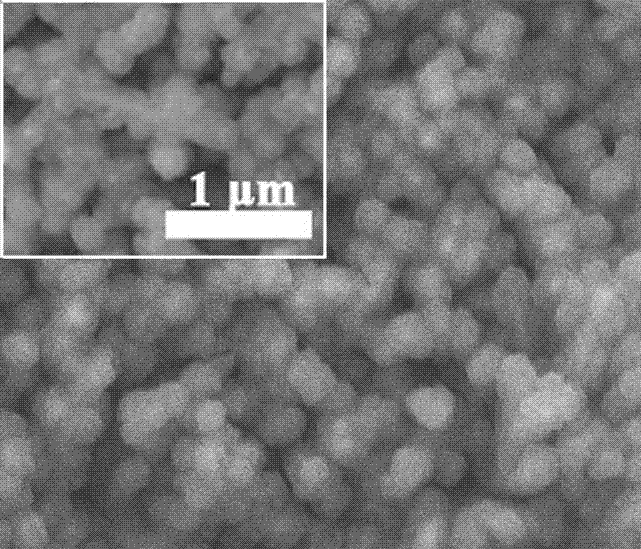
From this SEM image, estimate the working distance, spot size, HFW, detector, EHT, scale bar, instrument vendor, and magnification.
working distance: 7.8 mm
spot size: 2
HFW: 7.46 µm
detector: ETD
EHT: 8 kV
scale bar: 2000 nm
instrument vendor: FEI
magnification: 20 K X